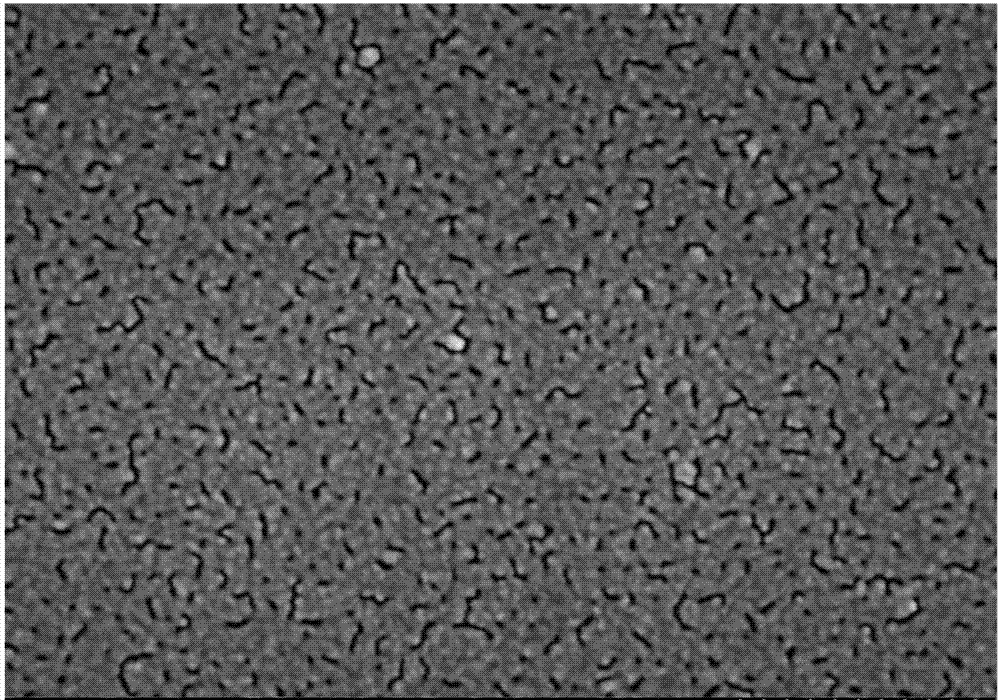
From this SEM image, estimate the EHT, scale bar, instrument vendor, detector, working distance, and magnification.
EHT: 5 kV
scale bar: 500 nm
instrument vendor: Hitachi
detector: SE(U)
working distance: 9 mm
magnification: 60 K X